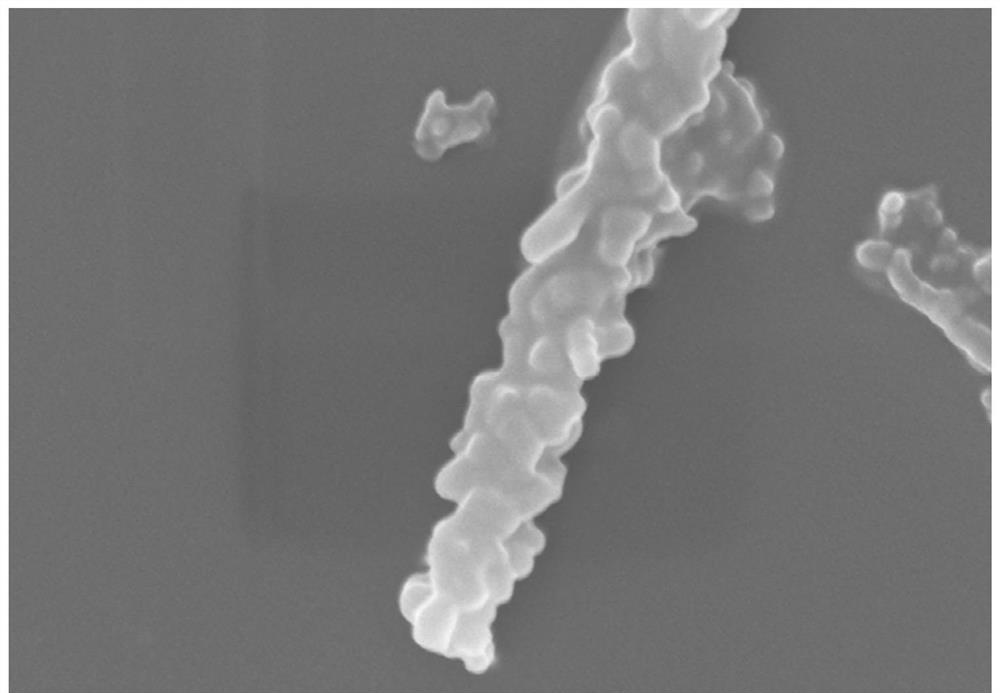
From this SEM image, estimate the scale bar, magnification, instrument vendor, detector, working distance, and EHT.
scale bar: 1000 nm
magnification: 40 K X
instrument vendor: Hitachi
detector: SE(M)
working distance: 6.9 mm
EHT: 5 kV